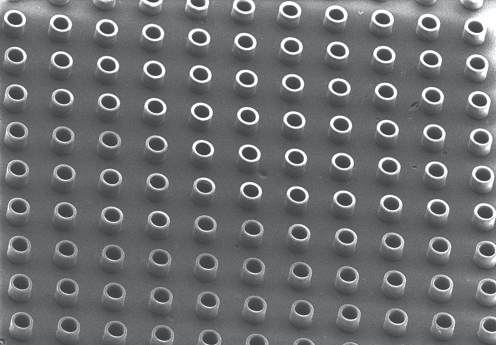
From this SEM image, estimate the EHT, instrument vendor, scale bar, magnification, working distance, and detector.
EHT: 10 kV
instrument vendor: Hitachi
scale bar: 300000 nm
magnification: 0.15 K X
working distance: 13.8 mm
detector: SE(U)